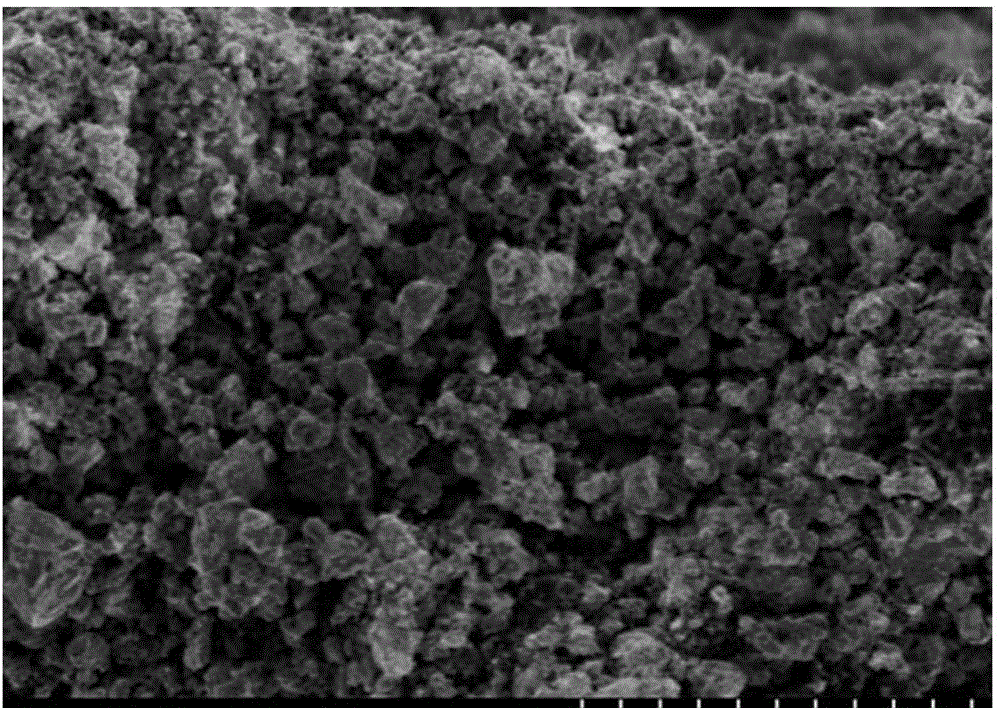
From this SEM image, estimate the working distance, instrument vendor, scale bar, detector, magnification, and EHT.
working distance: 4.5 mm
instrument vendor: Hitachi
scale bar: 10000 nm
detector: SE(M)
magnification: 5 K X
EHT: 2 kV